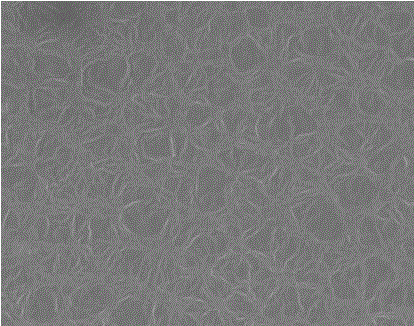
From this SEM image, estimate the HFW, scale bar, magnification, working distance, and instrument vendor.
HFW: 32 µm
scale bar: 10000 nm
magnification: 10.053 K X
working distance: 18.3 mm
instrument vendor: FEI